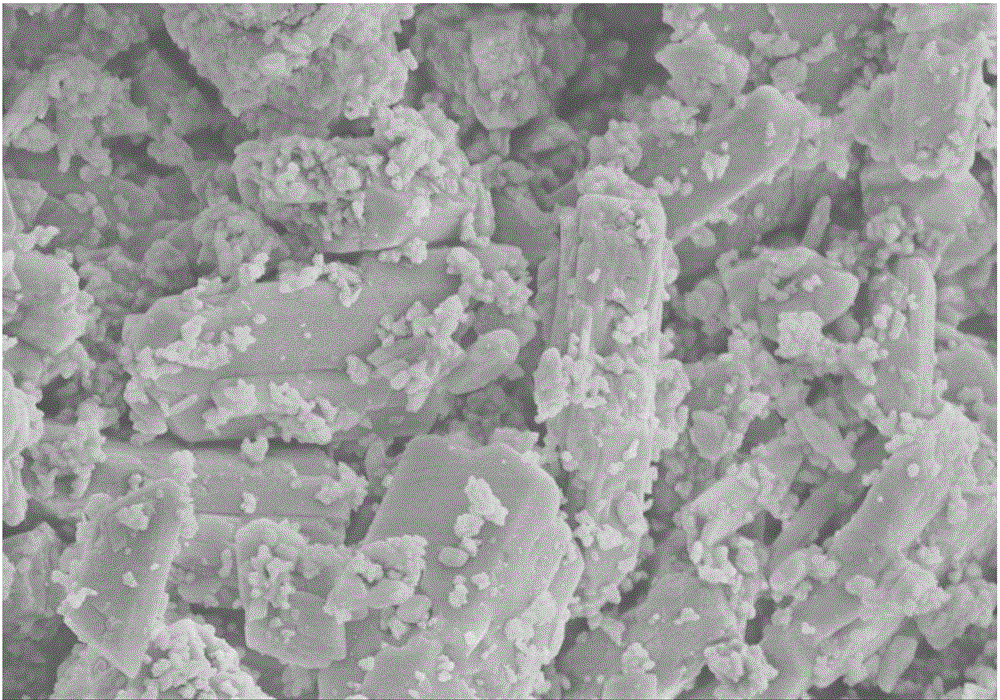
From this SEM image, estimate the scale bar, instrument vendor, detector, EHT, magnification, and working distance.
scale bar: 5000 nm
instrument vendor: Hitachi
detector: SE(M)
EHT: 5 kV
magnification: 10 K X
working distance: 13.4 mm